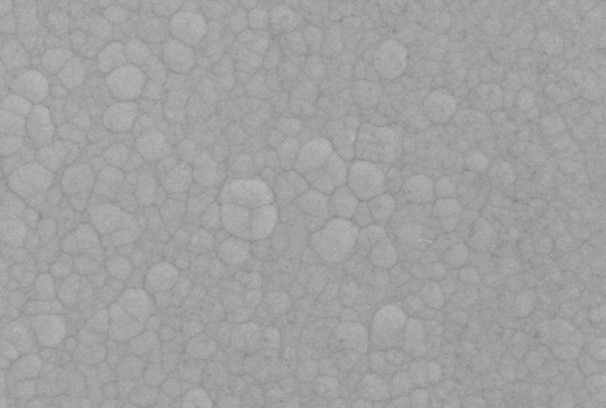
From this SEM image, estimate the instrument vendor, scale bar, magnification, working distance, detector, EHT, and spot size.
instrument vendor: JEOL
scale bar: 5000 nm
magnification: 3 K X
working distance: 10 mm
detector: SEI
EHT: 15 kV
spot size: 40